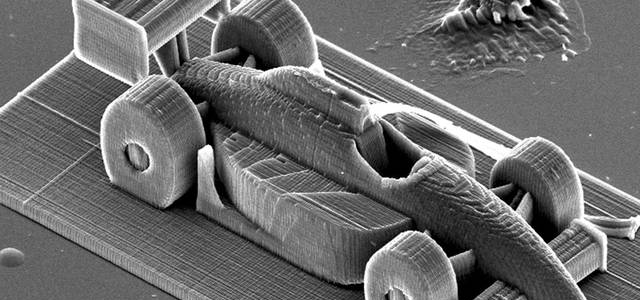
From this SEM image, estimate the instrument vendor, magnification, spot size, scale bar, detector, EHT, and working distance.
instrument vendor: FEI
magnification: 0.364 K X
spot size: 4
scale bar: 100000 nm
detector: SE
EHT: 20 kV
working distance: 27.2 mm